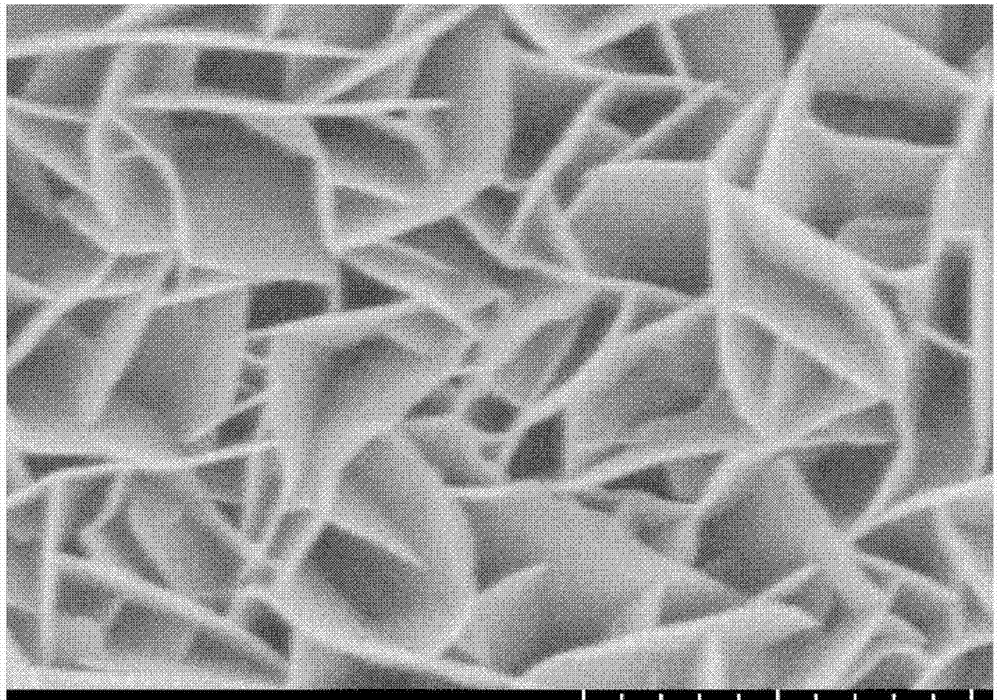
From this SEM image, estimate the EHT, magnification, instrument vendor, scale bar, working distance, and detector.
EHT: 5 kV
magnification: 50 K X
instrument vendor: Hitachi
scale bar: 1000 nm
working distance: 8.2 mm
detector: SE(UL)